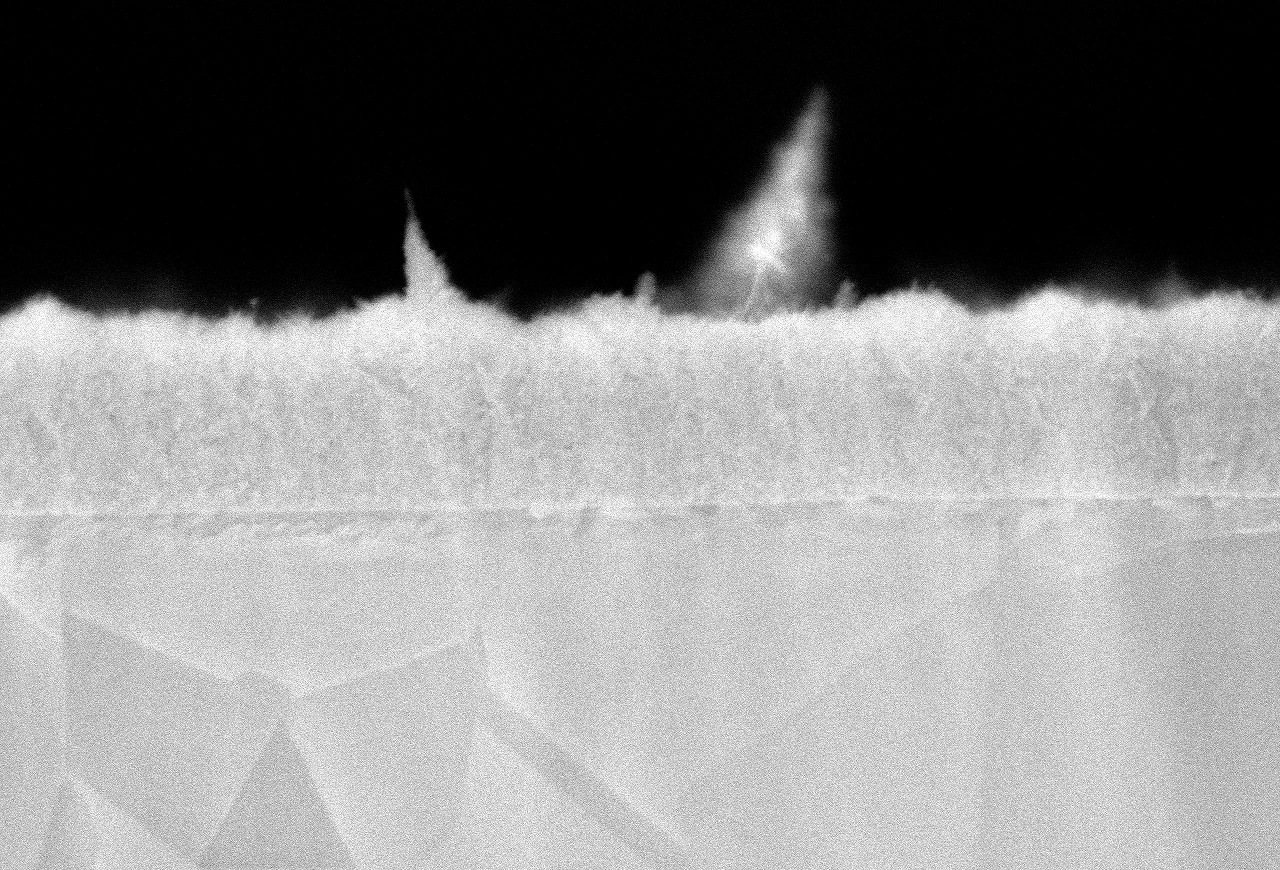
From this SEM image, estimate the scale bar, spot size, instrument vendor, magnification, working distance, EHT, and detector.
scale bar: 1000 nm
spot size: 40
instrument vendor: JEOL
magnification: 10 K X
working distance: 10 mm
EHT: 20 kV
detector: SEI